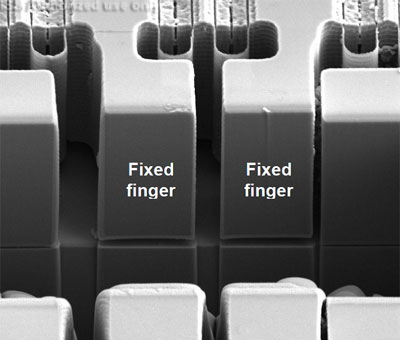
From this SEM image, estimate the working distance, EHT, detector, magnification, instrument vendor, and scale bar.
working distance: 4.3 mm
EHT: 5 kV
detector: ETD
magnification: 10 K X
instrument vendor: FEI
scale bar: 10000 nm